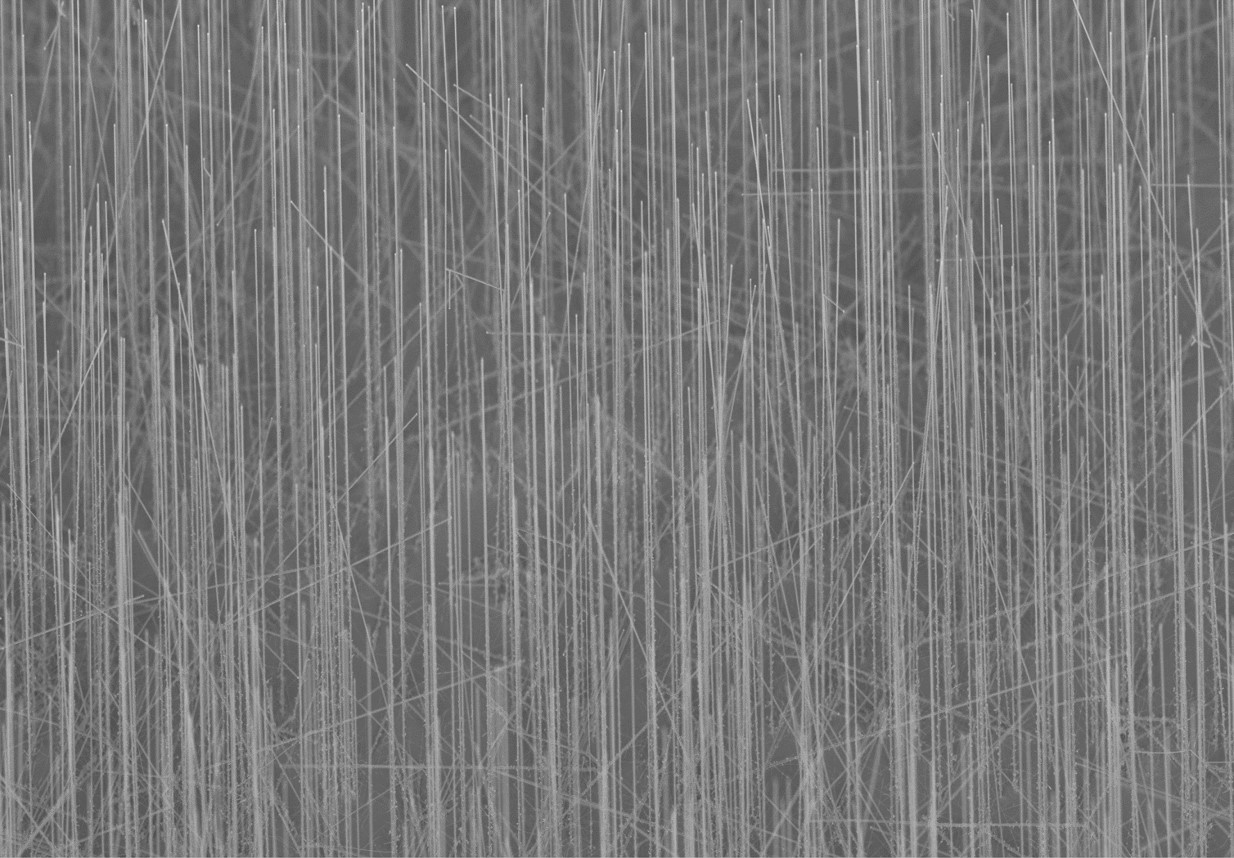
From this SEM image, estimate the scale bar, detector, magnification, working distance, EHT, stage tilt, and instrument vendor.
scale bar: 2000 nm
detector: InLens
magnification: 4 K X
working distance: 5.1 mm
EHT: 2 kV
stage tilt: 45°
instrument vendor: Zeiss